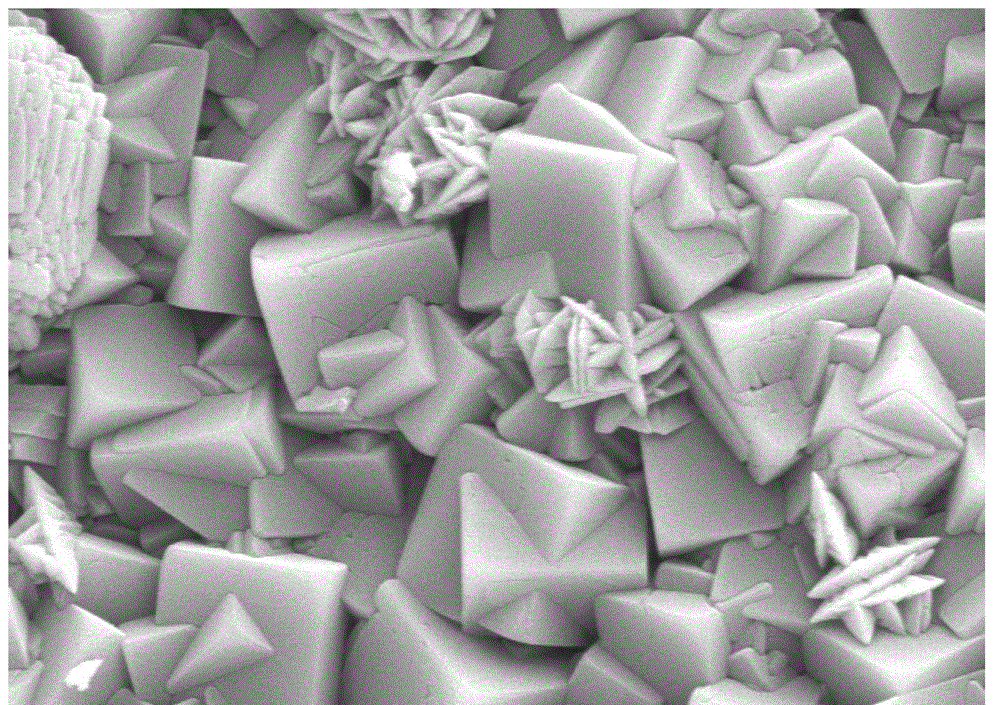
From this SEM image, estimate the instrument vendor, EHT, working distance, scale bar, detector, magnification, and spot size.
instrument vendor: FEI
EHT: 10 kV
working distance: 14.2 mm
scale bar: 2000 nm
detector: SE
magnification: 10 K X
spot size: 3